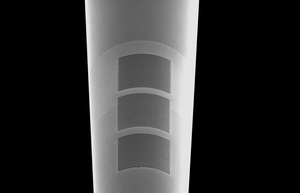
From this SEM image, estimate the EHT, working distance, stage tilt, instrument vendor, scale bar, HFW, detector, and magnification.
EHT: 5 kV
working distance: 4.1 mm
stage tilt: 52°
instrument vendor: FEI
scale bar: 60000 nm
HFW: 173 µm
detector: ETD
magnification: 2.4 K X